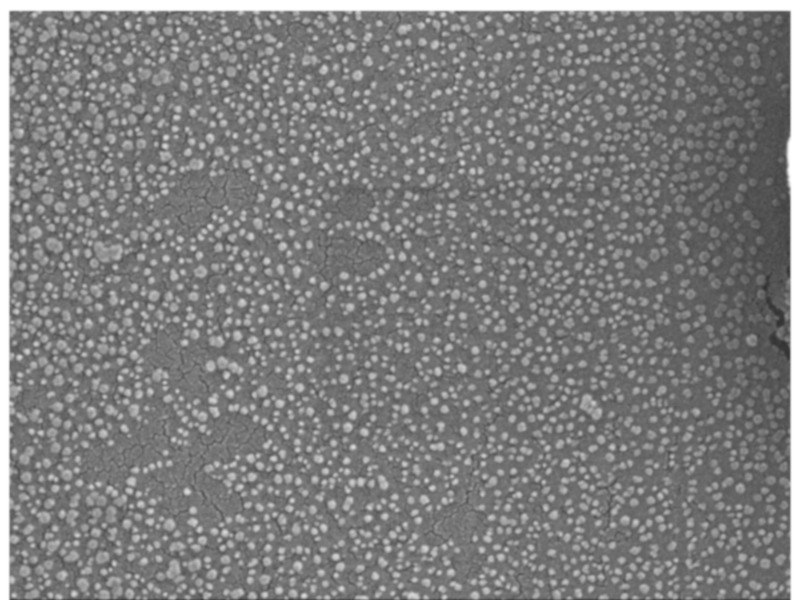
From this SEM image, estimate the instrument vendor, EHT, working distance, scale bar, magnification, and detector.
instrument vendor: JEOL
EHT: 10 kV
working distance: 6.4 mm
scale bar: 100 nm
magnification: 50 K X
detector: SEI